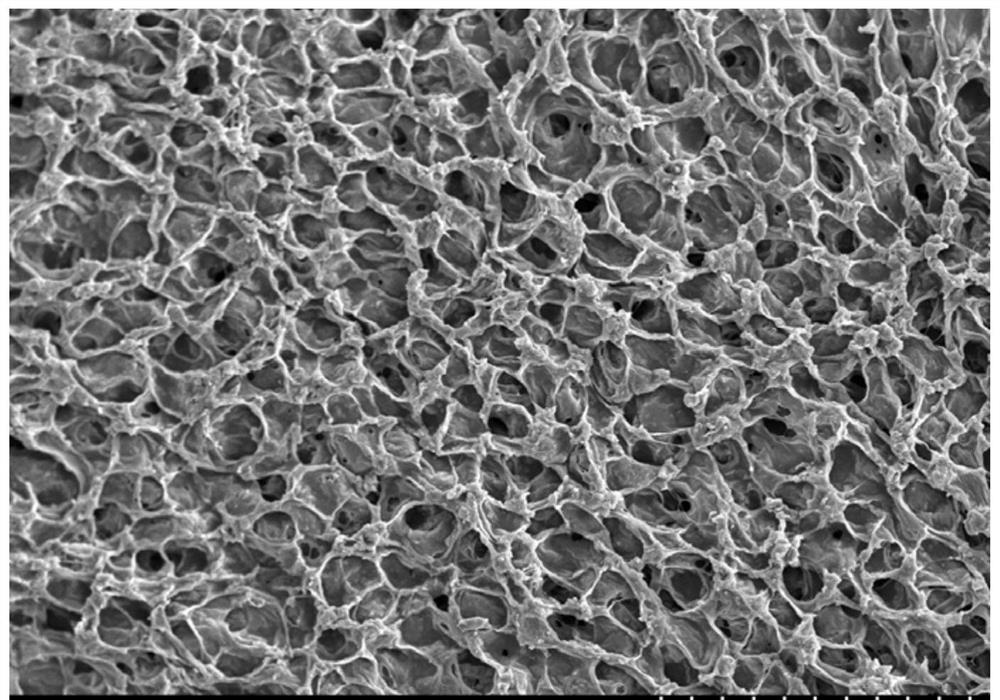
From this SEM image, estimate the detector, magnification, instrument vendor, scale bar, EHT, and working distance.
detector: SE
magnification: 0.2 K X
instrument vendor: Hitachi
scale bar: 200000 nm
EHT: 15 kV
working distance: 6.4 mm